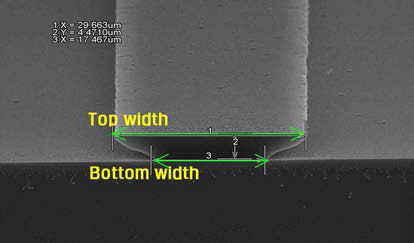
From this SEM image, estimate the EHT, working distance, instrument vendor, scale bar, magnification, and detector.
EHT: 15 kV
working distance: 11.7 mm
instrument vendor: Hitachi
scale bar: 20000 nm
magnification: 2 K X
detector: SE(U)